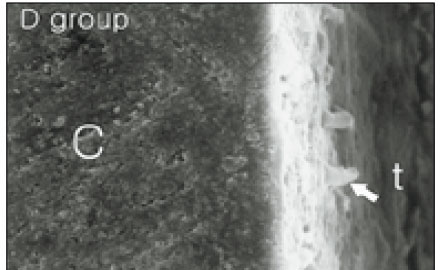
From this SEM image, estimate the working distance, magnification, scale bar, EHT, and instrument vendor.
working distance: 39 mm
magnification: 2 K X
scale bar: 10000 nm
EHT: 20 kV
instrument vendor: JEOL